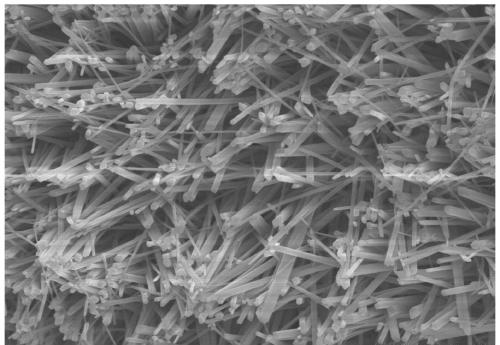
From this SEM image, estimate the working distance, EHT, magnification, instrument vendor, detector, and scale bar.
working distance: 9 mm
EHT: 15 kV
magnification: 3 K X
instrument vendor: Hitachi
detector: SE(UL)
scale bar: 10000 nm